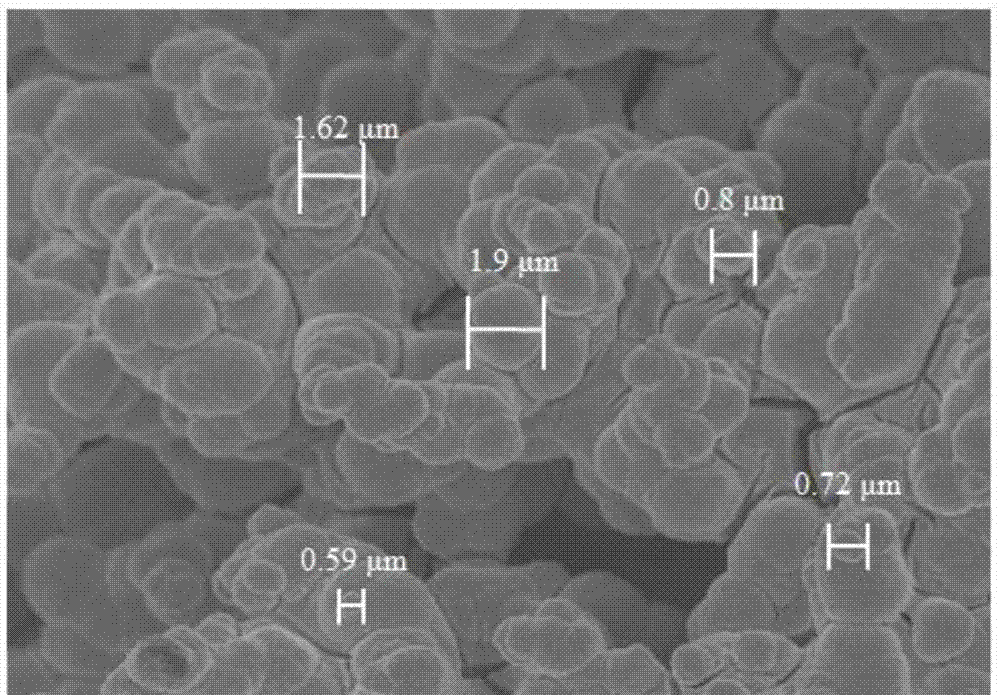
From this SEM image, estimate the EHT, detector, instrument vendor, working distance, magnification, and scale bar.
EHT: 5 kV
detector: SE(M)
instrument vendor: Hitachi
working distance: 5.3 mm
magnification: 5 K X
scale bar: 10000 nm